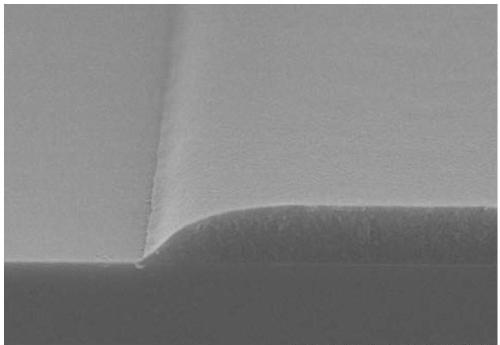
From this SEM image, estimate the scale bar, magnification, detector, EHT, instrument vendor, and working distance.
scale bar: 10000 nm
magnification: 5 K X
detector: SE(UL)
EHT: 15 kV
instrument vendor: Hitachi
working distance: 7 mm